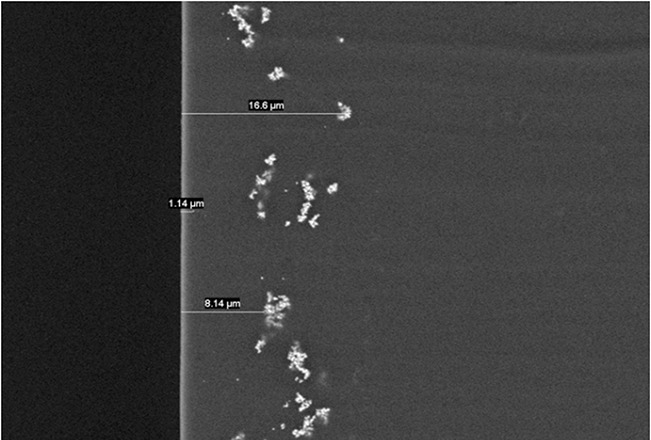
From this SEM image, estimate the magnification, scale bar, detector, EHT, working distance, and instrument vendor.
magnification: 2 K X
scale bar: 20000 nm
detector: BSE3D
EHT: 10 kV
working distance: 9.3 mm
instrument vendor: Hitachi